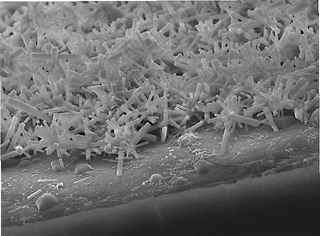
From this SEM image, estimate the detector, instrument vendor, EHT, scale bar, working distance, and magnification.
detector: SEI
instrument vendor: JEOL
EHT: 15 kV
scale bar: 10000 nm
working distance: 8.8 mm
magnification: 2.7 K X